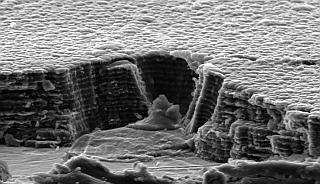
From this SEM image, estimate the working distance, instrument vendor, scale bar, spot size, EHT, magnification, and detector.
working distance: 12.4 mm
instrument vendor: FEI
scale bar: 5000 nm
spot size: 2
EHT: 5 kV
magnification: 11.349 K X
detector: SE